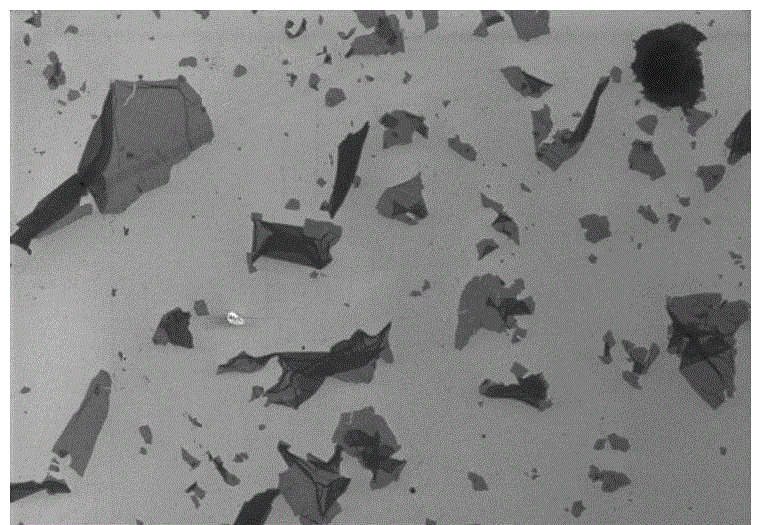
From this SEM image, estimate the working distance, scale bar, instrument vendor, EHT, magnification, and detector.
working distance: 8.9 mm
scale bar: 100000 nm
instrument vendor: Hitachi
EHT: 3 kV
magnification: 0.4 K X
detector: SE(M)